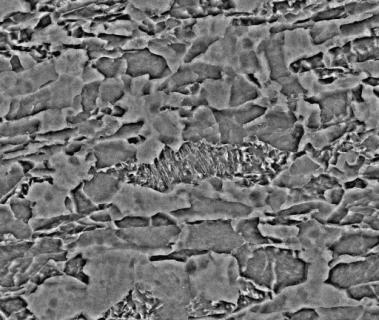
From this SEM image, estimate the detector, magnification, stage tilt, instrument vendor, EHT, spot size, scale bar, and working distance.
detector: BSED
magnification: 5 K X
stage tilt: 0°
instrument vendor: FEI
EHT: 25 kV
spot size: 3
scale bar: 20000 nm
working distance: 10.2 mm